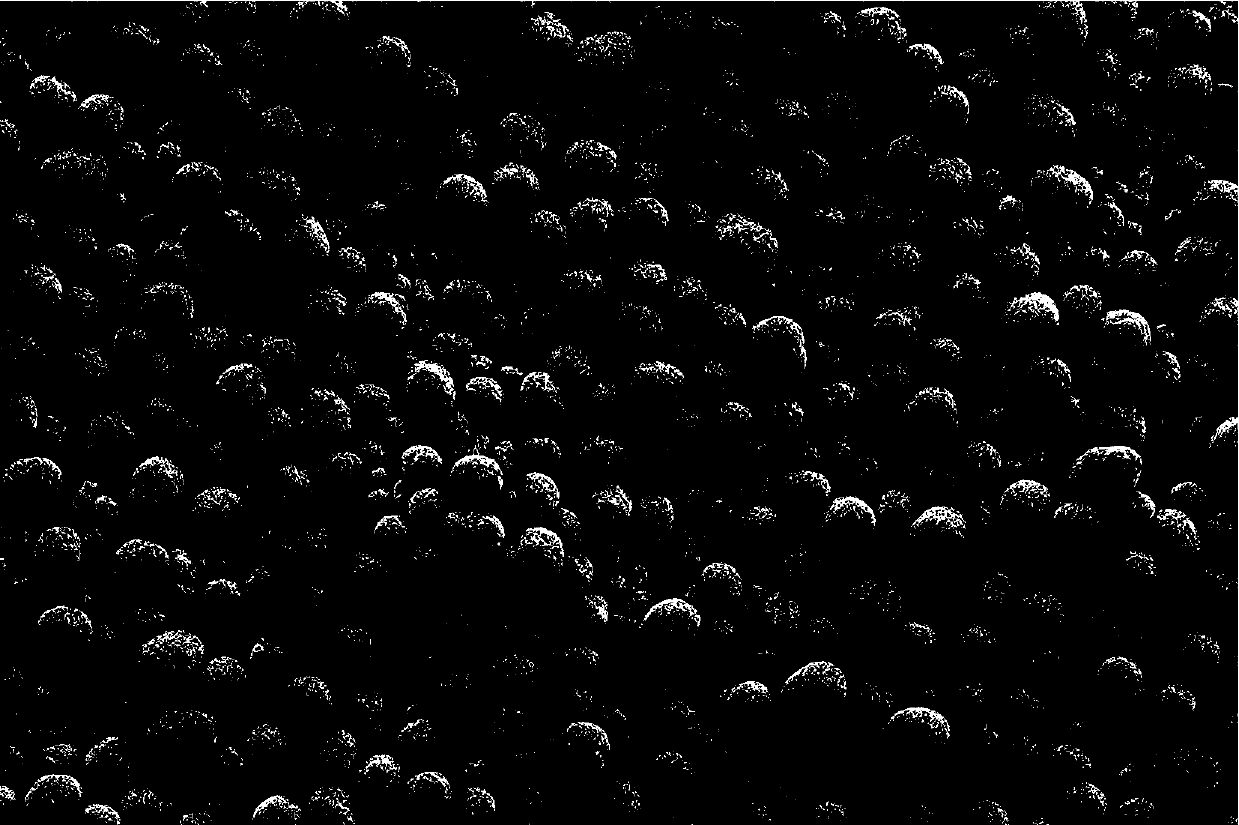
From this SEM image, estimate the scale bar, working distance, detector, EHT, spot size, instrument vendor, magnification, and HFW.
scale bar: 50000 nm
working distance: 11.6 mm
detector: ETD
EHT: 6 kV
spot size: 7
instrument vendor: Thermo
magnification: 1 K X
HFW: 207 µm